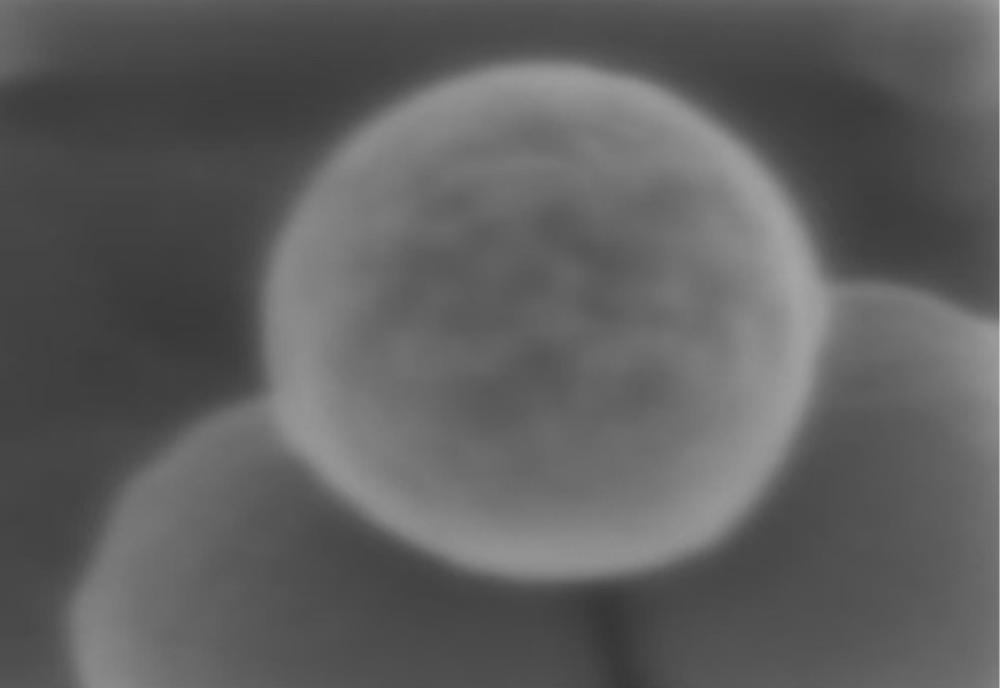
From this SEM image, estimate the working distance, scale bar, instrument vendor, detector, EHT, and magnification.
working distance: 1.5 mm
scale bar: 20 nm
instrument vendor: Zeiss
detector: InLens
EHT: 0.5 kV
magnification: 350 K X